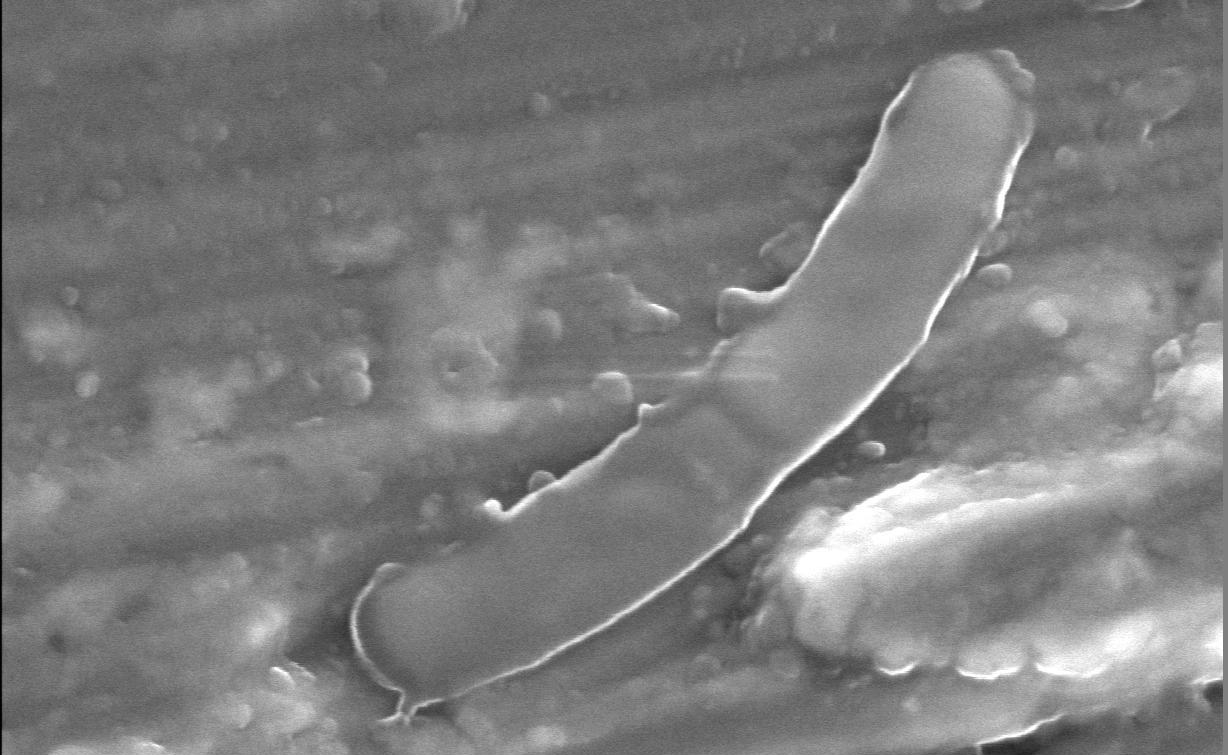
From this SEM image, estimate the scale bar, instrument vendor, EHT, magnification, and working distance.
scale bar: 1000 nm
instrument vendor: JEOL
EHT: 7 kV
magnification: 20 K X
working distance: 11 mm